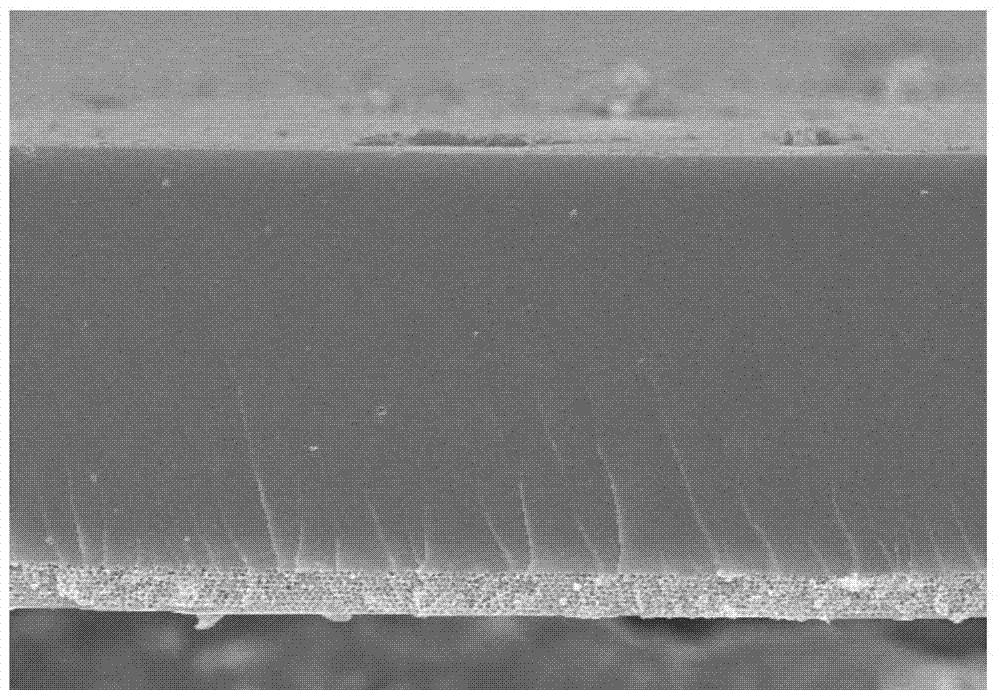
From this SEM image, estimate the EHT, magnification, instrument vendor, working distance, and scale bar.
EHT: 10 kV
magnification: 3.5 K X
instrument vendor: Hitachi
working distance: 8.5 mm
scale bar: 10000 nm